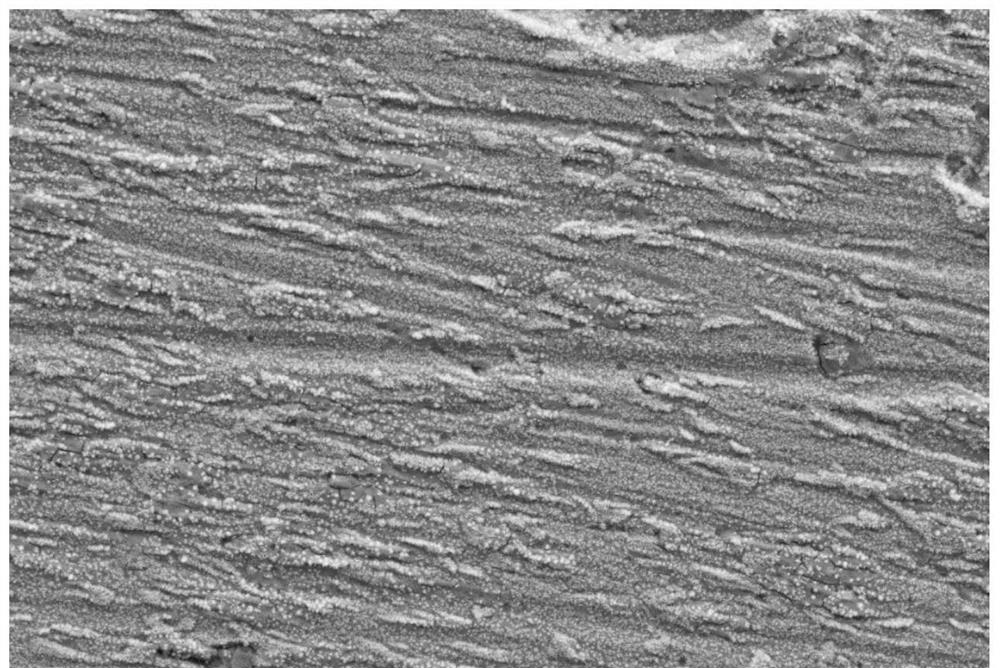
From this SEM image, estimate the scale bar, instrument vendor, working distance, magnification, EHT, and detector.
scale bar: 10000 nm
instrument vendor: Zeiss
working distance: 9.8 mm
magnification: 1 K X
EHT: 20 kV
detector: SE2